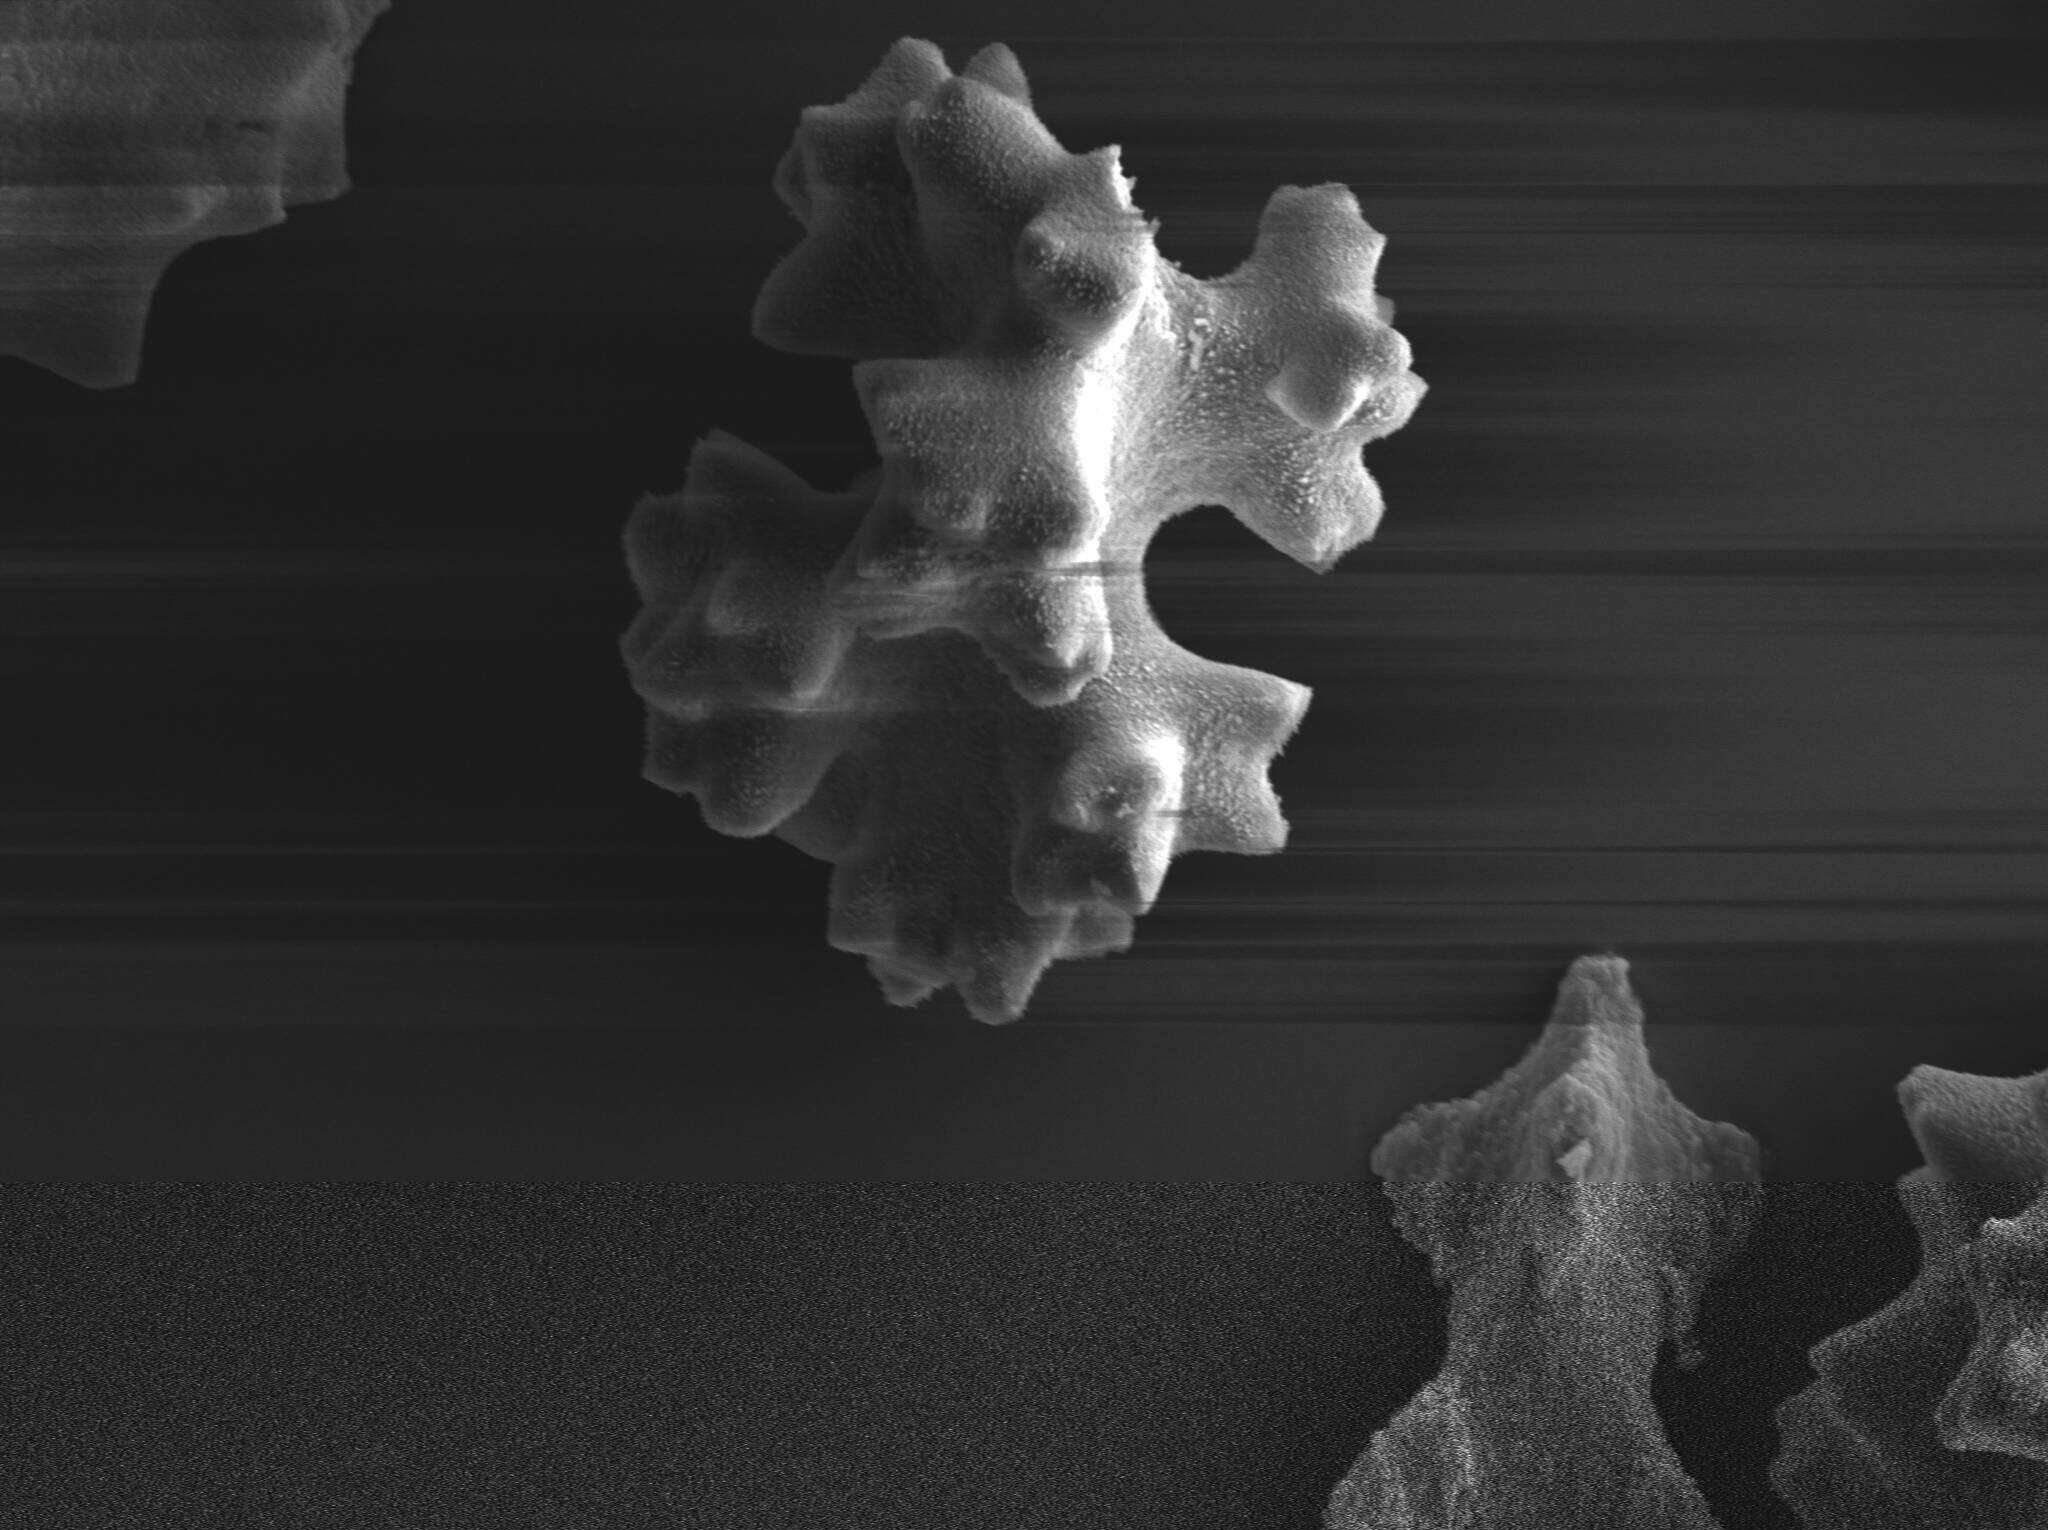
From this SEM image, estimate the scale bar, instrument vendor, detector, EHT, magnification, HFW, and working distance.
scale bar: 20000 nm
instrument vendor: Zeiss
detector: SE1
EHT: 12 kV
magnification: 1 K X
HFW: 114.4 µm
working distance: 12.7 mm